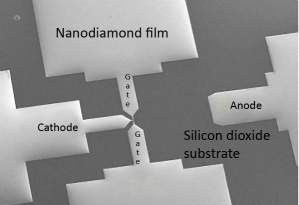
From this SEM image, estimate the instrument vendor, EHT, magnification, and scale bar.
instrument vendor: JEOL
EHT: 20 kV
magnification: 0.06 K X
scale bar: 500000 nm